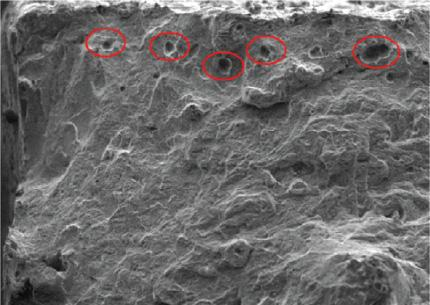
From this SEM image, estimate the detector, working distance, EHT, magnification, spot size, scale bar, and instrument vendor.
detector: SE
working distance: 12.7 mm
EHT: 10 kV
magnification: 0.101 K X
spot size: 3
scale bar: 200000 nm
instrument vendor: FEI